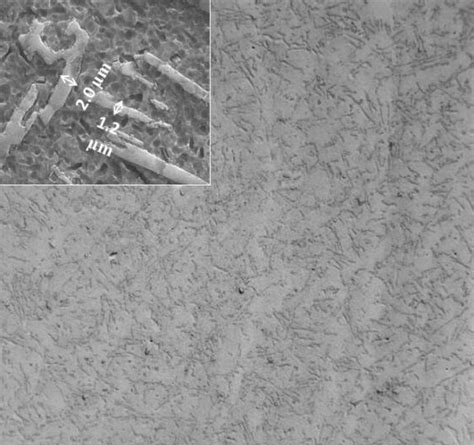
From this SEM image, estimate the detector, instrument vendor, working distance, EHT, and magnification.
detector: SEI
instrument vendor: JEOL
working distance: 10 mm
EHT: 20 kV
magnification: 0.1 K X